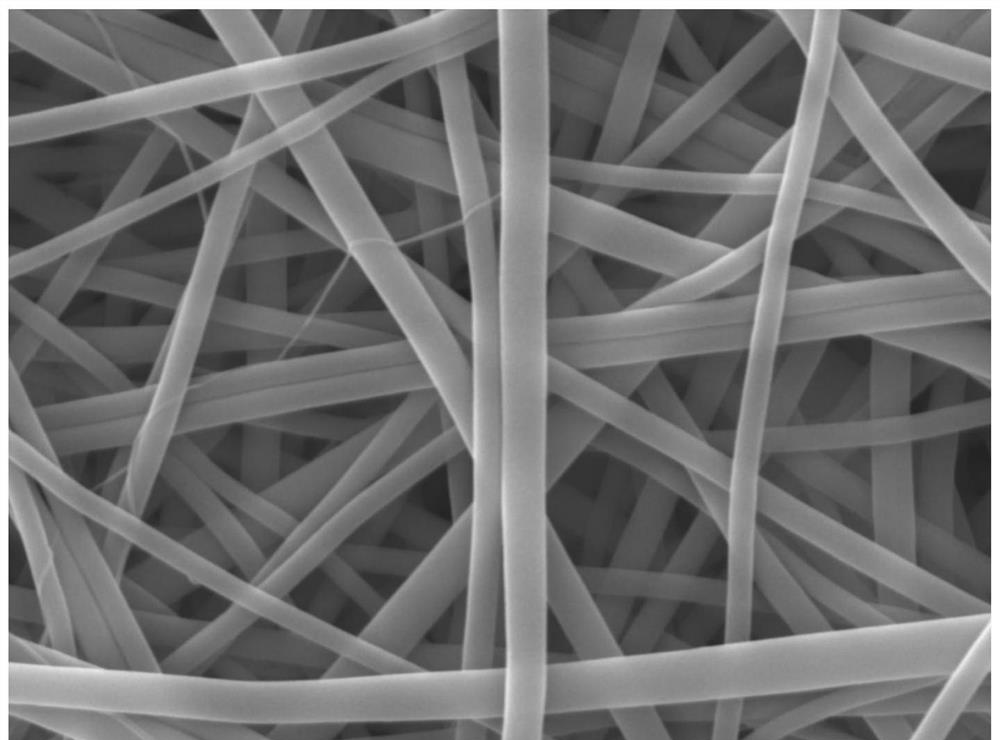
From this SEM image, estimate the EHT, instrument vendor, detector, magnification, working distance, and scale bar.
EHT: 10 kV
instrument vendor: JEOL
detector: LED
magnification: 20 K X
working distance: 10.7 mm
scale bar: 1000 nm